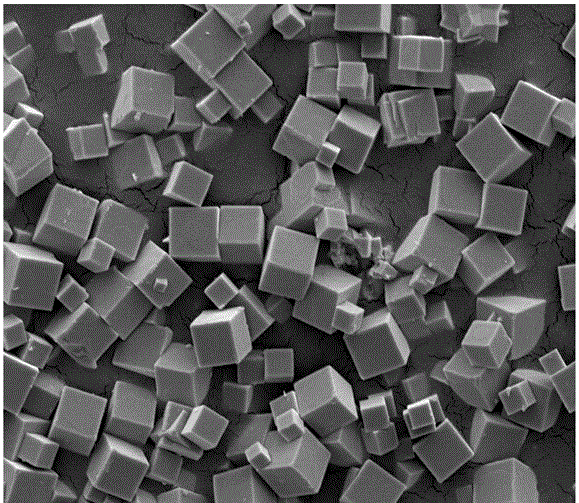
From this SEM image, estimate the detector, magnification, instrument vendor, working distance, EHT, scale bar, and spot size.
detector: ETD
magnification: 20 K X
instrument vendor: FEI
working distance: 9.1 mm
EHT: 5 kV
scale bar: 5000 nm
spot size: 3